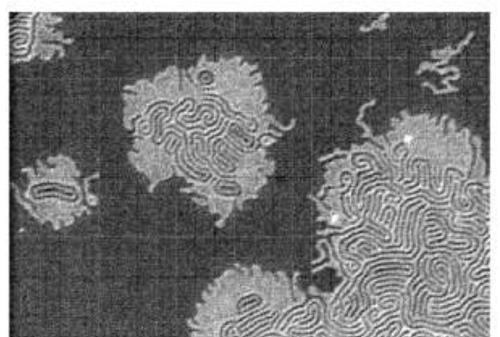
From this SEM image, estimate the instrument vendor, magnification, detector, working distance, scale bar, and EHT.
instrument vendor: Hitachi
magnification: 50 K X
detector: SE(M)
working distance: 4.4 mm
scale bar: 1000 nm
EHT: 2 kV